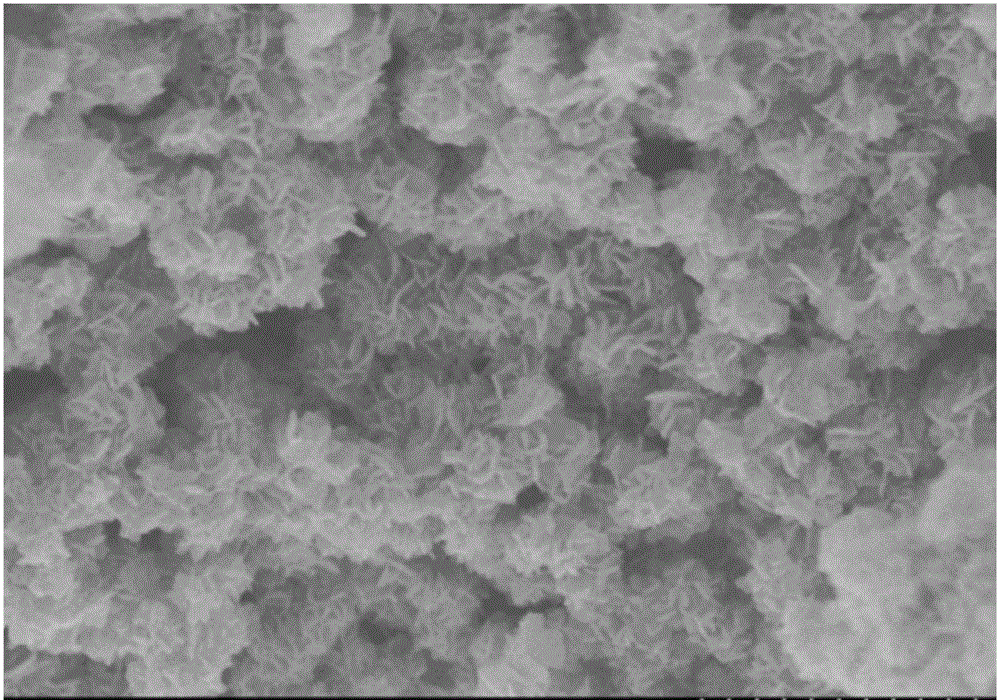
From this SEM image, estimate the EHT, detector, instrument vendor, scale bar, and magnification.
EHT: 4 kV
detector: SE(U)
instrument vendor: Hitachi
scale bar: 1000 nm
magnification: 35 K X